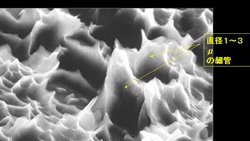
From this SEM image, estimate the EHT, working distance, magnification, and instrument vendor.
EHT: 15 kV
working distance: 24 mm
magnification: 20 K X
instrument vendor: JEOL